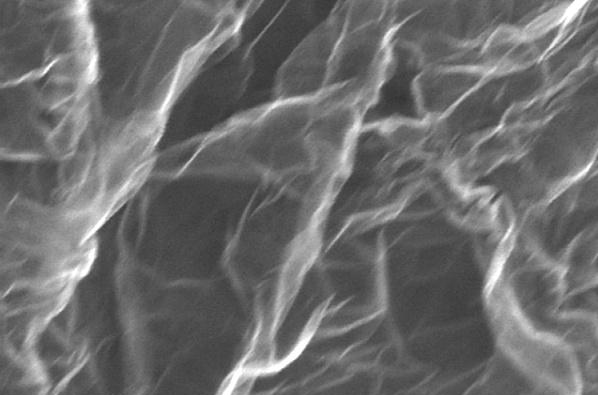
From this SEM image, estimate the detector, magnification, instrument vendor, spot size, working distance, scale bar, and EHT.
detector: TLD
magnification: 160 K X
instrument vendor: FEI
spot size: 4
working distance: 5.3 mm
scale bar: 500 nm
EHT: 5 kV